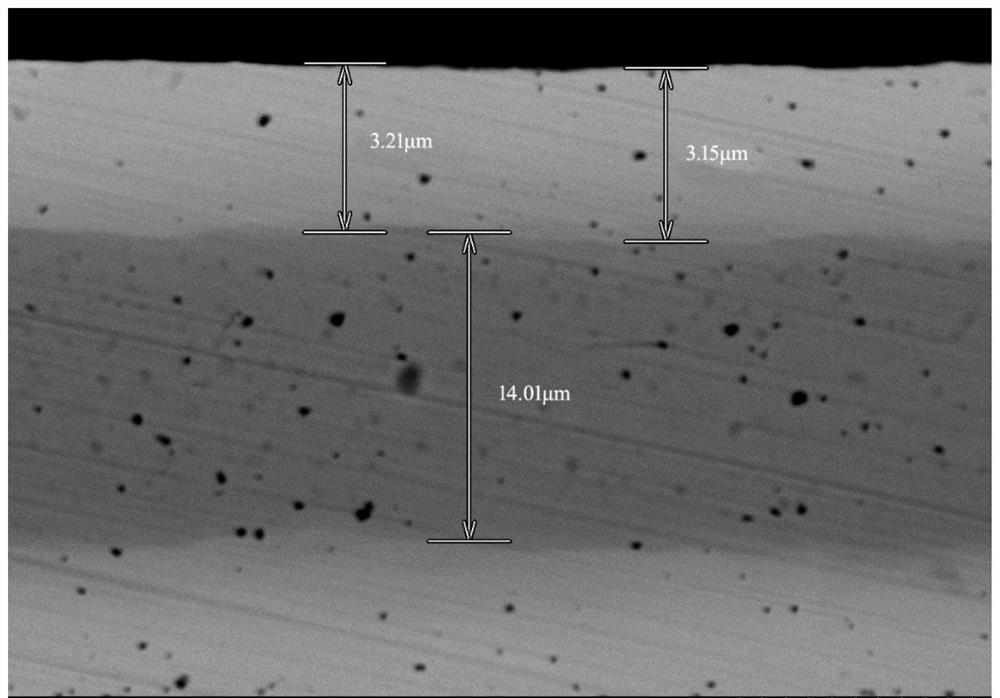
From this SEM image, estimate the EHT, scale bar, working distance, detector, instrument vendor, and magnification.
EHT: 15 kV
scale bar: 10000 nm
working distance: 14.4 mm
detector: BSECOMP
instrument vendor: Hitachi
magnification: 3 K X